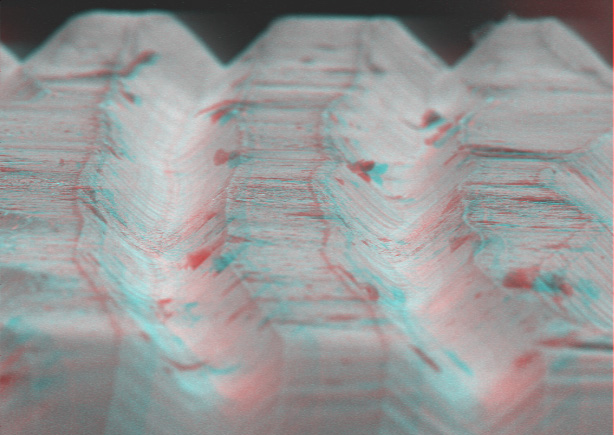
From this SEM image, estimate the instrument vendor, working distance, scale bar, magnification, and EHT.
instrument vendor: other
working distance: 30 mm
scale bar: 100000 nm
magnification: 0.39 K X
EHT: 15 kV